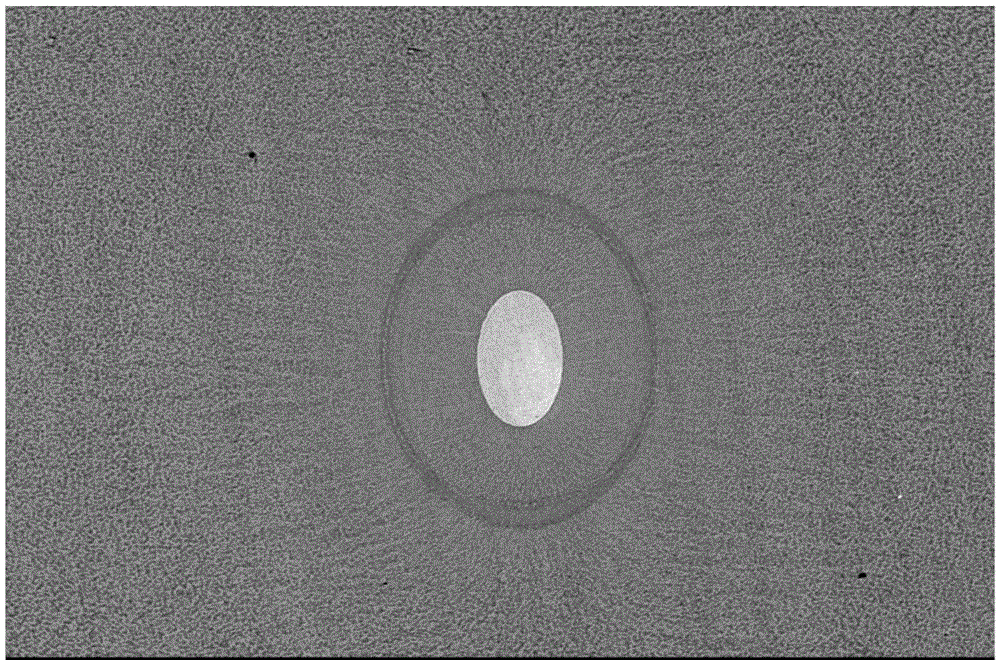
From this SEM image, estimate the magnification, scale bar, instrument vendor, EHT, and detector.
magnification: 0.27 K X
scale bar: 50000 nm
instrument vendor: JEOL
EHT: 20 kV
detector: SEI